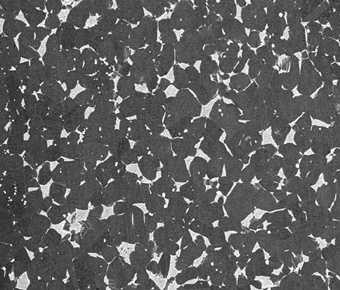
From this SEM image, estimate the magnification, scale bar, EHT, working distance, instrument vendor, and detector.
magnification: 0.5 K X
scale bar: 200000 nm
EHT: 20 kV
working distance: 14.9 mm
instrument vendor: FEI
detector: Z Cont